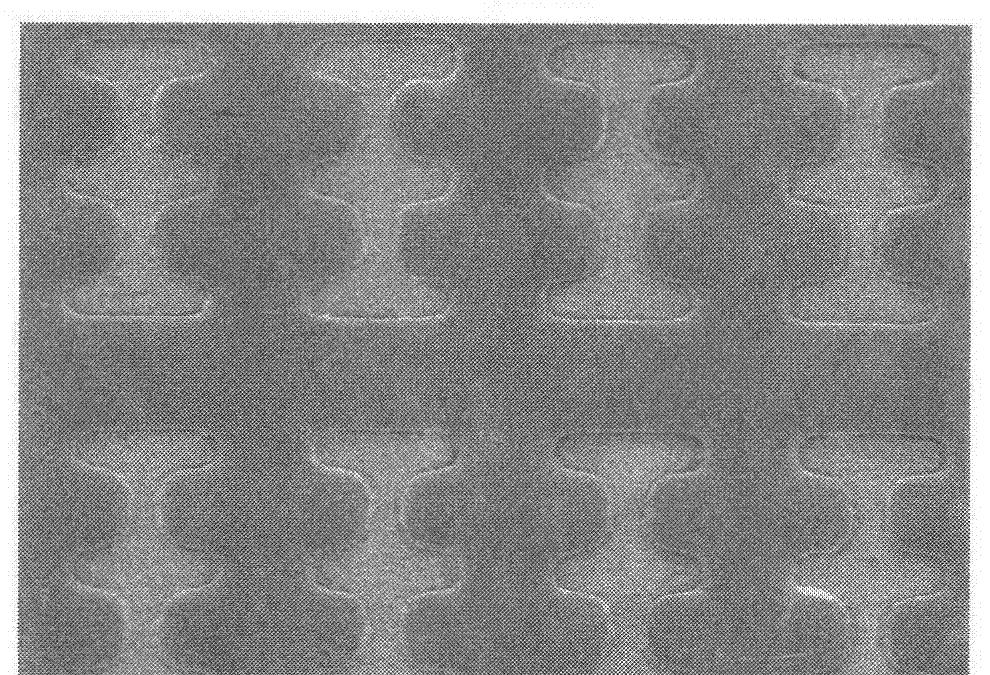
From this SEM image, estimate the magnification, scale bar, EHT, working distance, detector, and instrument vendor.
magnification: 0.2 K X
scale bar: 200000 nm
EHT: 2 kV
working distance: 7.6 mm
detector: SE(M)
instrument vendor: Hitachi